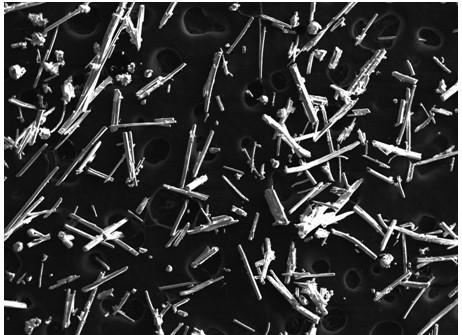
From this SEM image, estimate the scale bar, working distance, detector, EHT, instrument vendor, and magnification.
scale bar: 100000 nm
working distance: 11 mm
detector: SEI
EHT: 15 kV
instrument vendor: JEOL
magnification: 0.043 K X